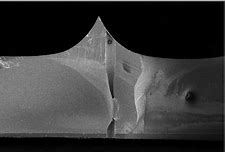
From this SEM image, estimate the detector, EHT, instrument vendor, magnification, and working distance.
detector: InLens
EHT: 5 kV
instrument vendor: Zeiss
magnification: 0.05 K X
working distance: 3.6 mm